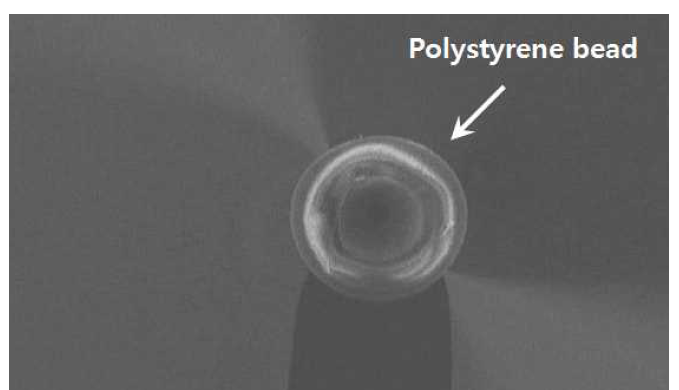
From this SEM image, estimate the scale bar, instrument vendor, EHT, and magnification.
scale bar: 10000 nm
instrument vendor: Hitachi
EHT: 5 kV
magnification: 0.75 K X